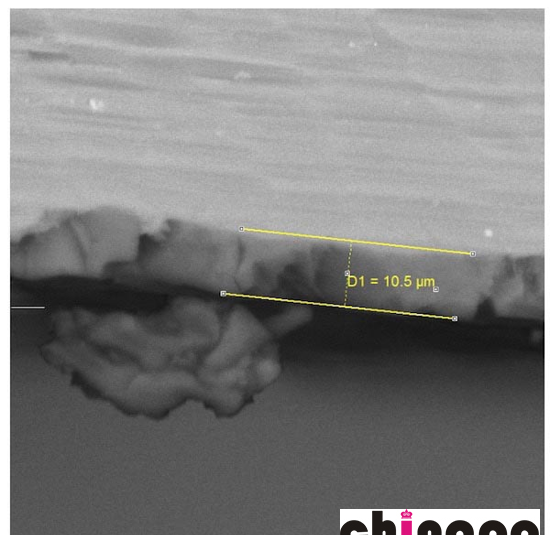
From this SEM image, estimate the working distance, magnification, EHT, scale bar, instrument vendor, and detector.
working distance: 25.94 mm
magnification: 2.34 K X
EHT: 25 kV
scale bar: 20000 nm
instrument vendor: FEI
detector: BSE